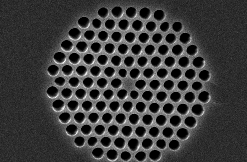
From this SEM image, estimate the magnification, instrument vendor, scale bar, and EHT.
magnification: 3 K X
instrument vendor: JEOL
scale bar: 5000 nm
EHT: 15 kV